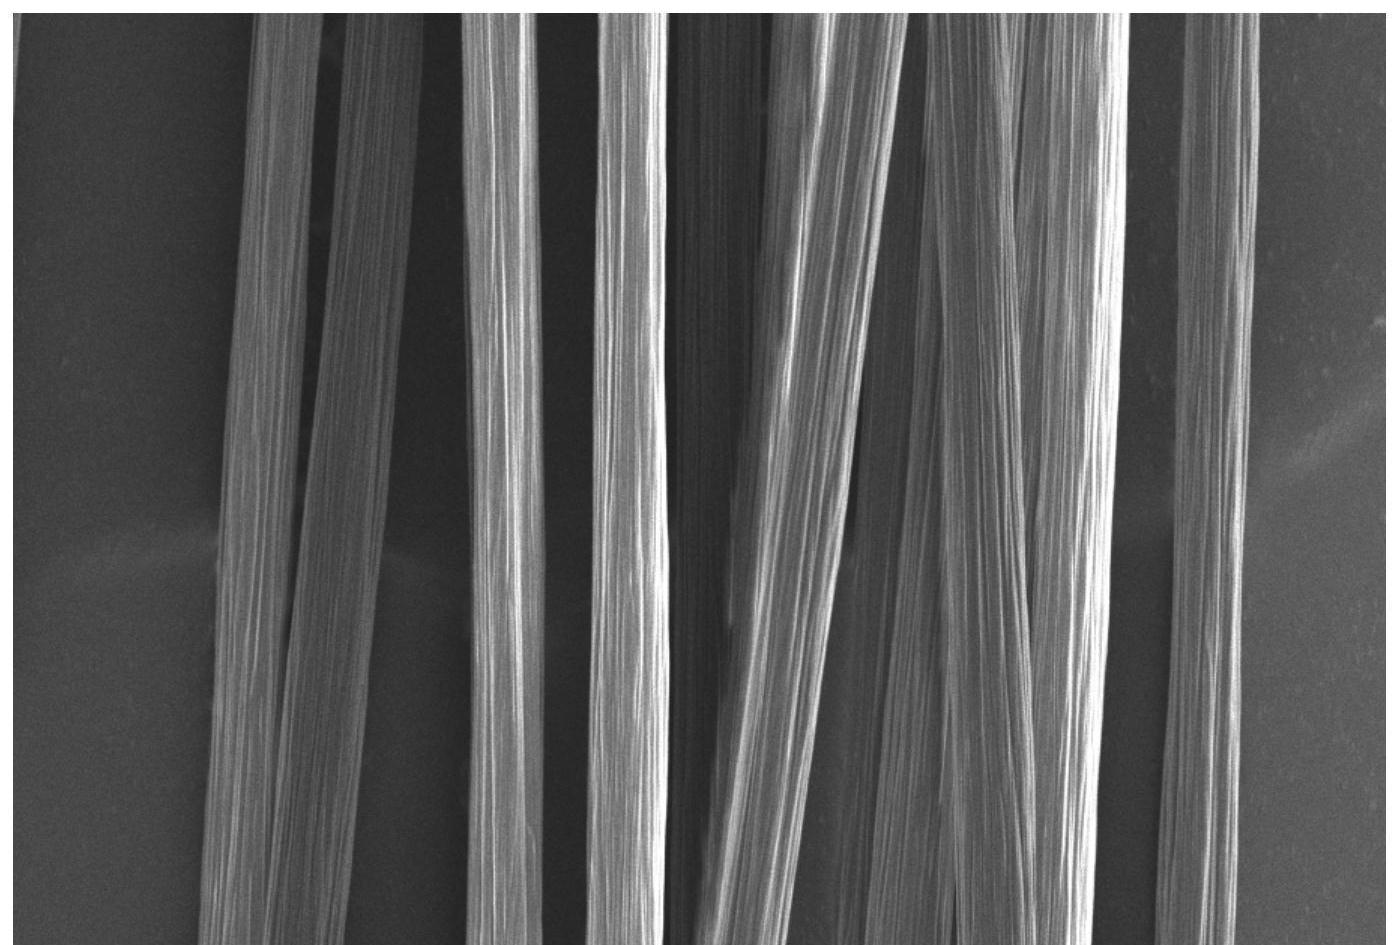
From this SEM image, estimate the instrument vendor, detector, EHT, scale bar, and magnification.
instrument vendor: JEOL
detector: SED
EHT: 3 kV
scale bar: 10000 nm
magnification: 1 K X